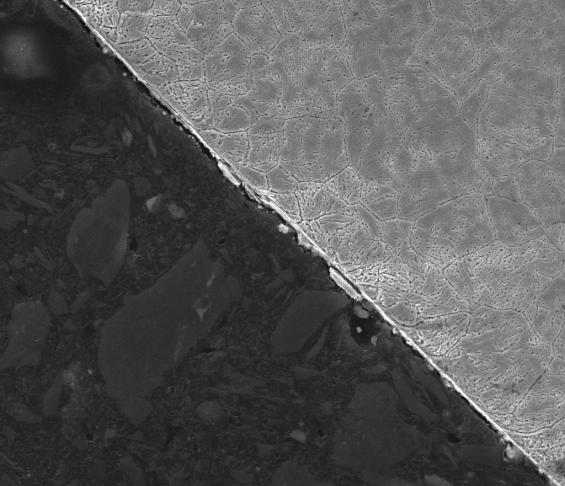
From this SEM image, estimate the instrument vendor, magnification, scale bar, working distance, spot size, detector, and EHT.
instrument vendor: FEI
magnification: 1.6 K X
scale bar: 50000 nm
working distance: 10 mm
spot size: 3.5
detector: ETD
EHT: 25 kV